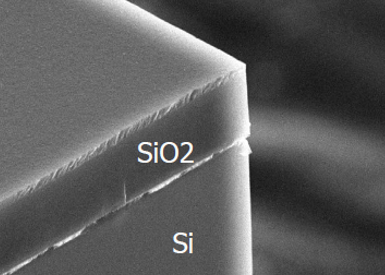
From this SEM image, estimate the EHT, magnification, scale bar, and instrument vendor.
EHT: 15 kV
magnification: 6 K X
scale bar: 2000 nm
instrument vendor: JEOL